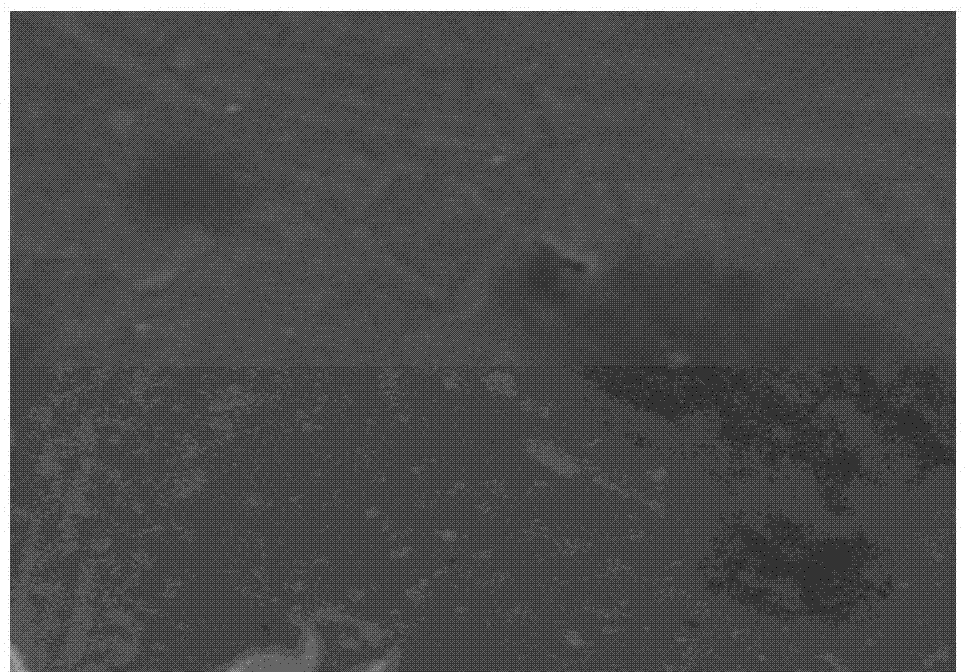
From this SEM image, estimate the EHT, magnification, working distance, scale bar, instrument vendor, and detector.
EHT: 3 kV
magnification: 50 K X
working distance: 8.1 mm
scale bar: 1000 nm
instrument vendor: Hitachi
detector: SE(M)